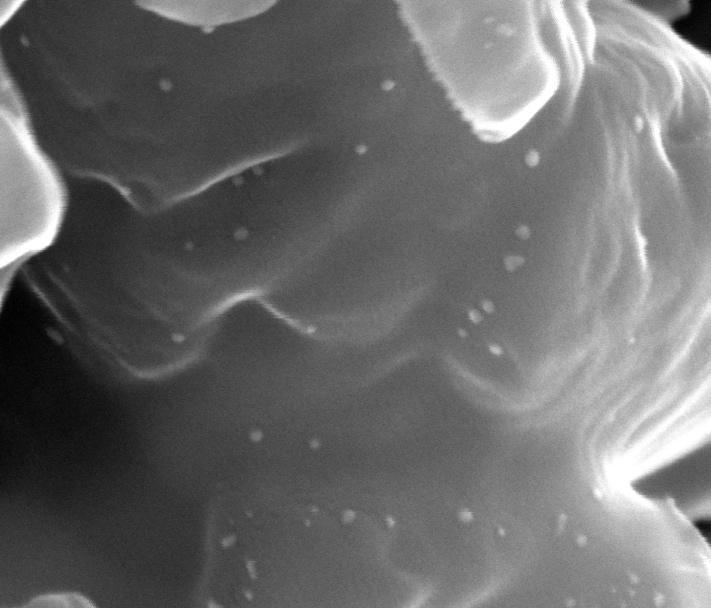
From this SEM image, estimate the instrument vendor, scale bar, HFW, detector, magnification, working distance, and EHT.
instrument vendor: FEI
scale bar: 200 nm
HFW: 0.853 µm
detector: SE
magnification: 300 K X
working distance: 8.9 mm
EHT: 30 kV